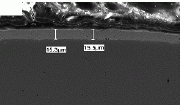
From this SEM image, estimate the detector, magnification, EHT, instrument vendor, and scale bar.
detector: SEI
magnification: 0.5 K X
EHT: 20 kV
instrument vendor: JEOL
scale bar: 50000 nm